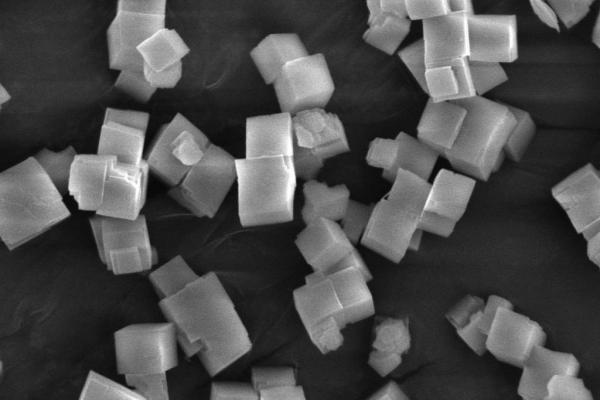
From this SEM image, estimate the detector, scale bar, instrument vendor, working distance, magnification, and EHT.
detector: SE1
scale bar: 2000 nm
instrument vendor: Zeiss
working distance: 8.5 mm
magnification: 5 K X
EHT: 10 kV